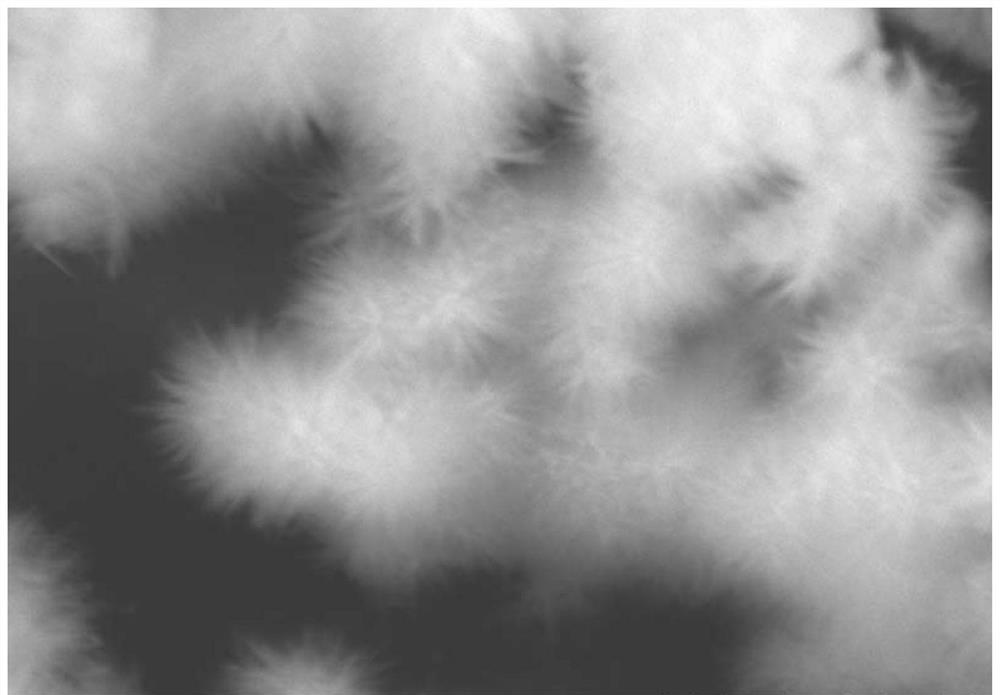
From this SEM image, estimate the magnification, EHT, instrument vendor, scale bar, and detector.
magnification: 8 K X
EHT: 20 kV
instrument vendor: Hitachi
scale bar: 5000 nm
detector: SE